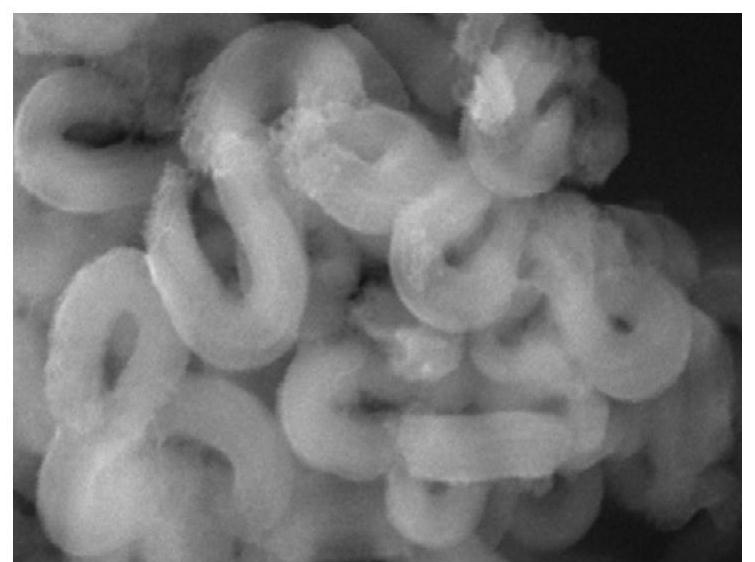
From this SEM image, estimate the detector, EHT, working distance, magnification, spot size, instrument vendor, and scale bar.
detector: SE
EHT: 15 kV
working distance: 8.2 mm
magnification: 40 K X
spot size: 3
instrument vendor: FEI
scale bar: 500 nm